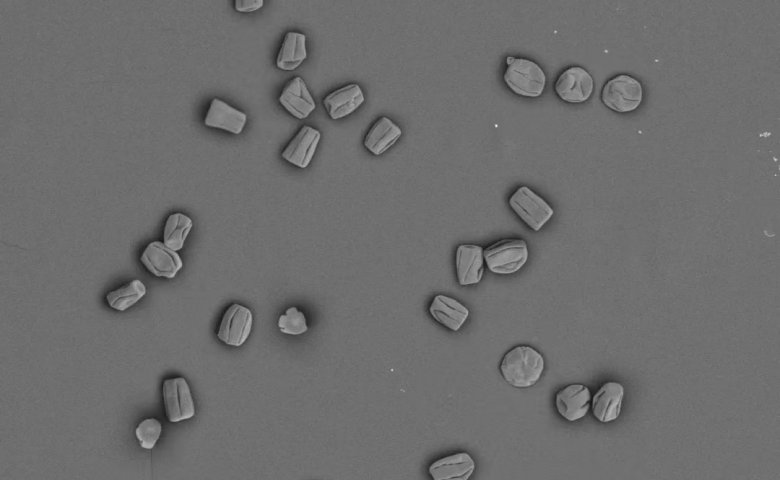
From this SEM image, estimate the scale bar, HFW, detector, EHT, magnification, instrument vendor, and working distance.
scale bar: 300000 nm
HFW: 1420 µm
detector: BSD Full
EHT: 10 kV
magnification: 0.36 K X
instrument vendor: Thermo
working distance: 10.491 mm